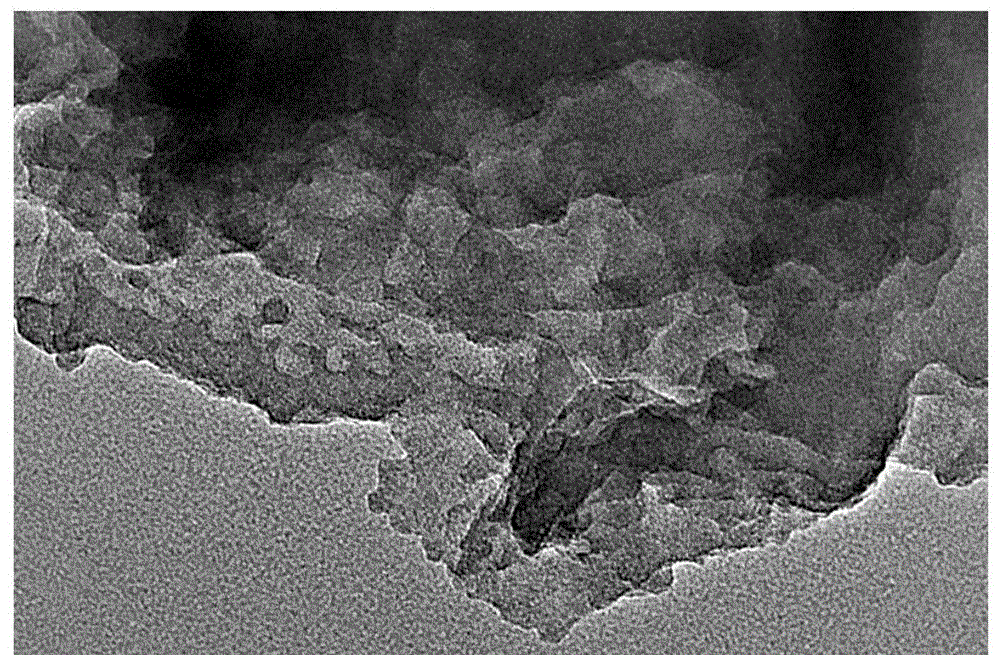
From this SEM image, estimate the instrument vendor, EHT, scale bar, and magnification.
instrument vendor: FEI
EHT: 200 kV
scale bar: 100 nm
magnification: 97 K X